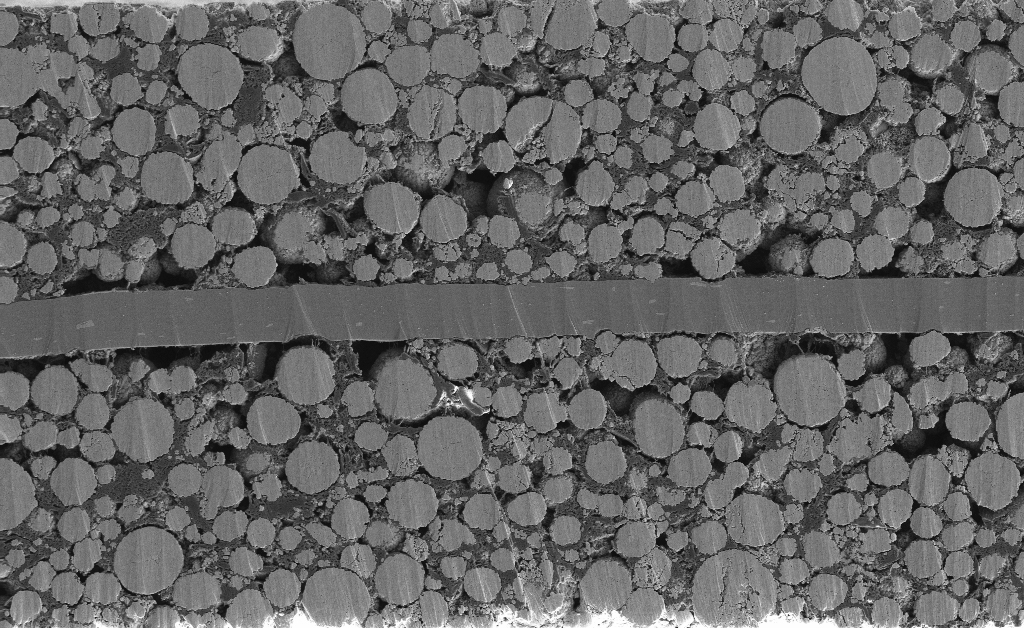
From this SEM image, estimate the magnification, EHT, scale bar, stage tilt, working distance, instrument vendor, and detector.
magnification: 0.5 K X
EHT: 5 kV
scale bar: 20000 nm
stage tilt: -2.3°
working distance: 5.3 mm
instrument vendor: Zeiss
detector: SE2